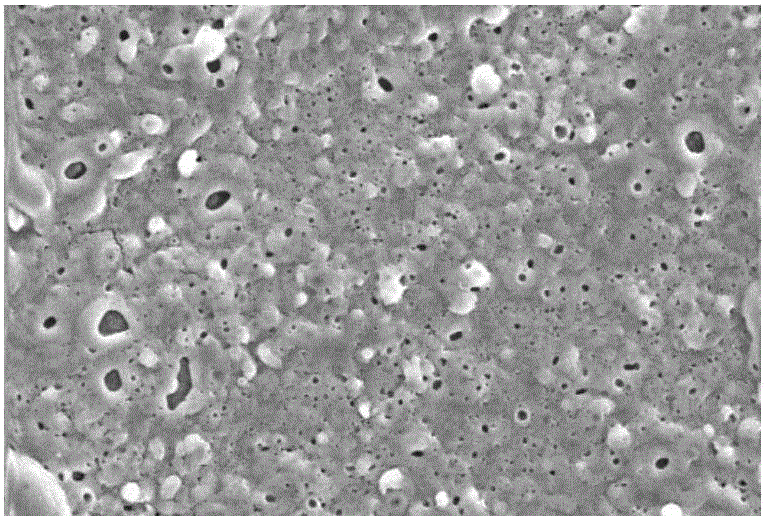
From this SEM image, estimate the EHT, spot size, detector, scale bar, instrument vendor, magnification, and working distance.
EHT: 20 kV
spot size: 3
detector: SE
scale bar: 20000 nm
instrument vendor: FEI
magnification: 2 K X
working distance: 6.6 mm